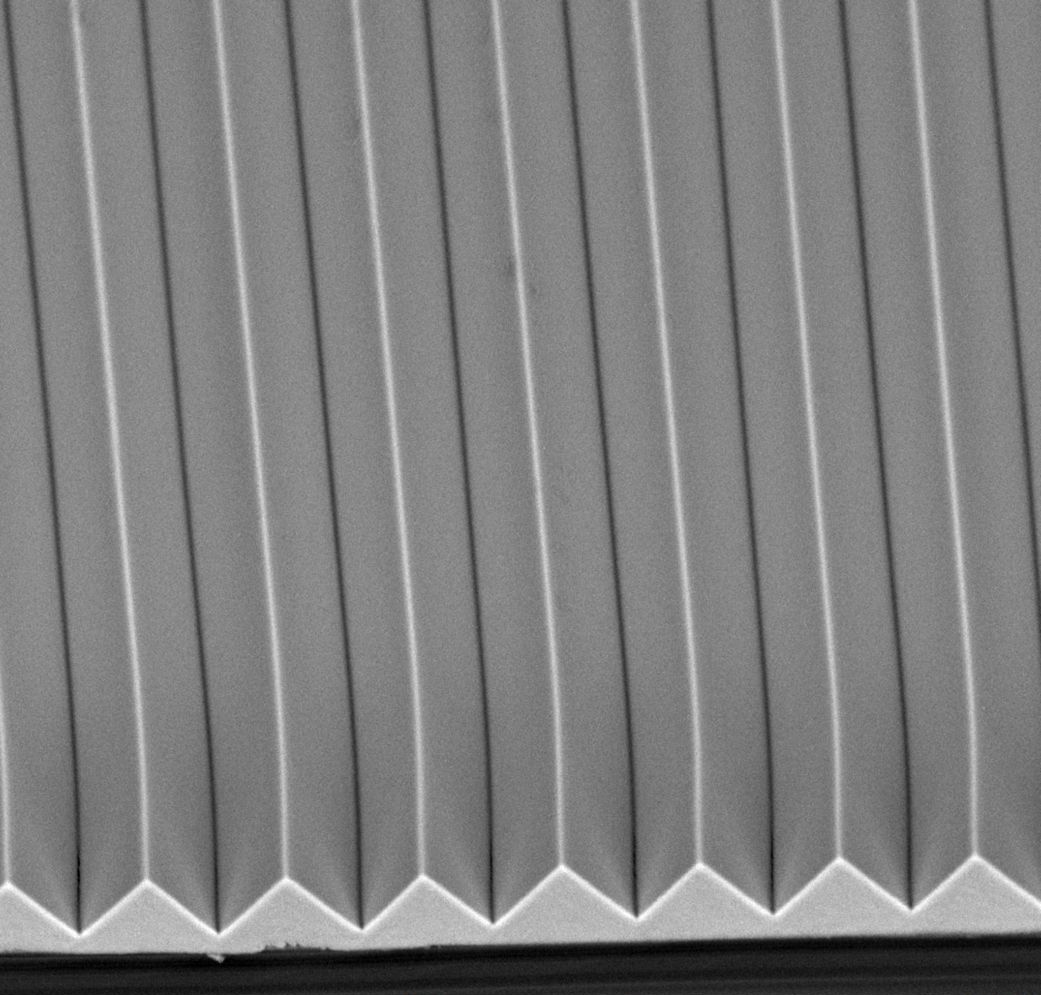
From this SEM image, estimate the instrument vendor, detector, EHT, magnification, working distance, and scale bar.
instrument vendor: other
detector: BSE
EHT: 15 kV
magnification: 1.5 K X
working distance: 8.1 mm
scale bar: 20000 nm